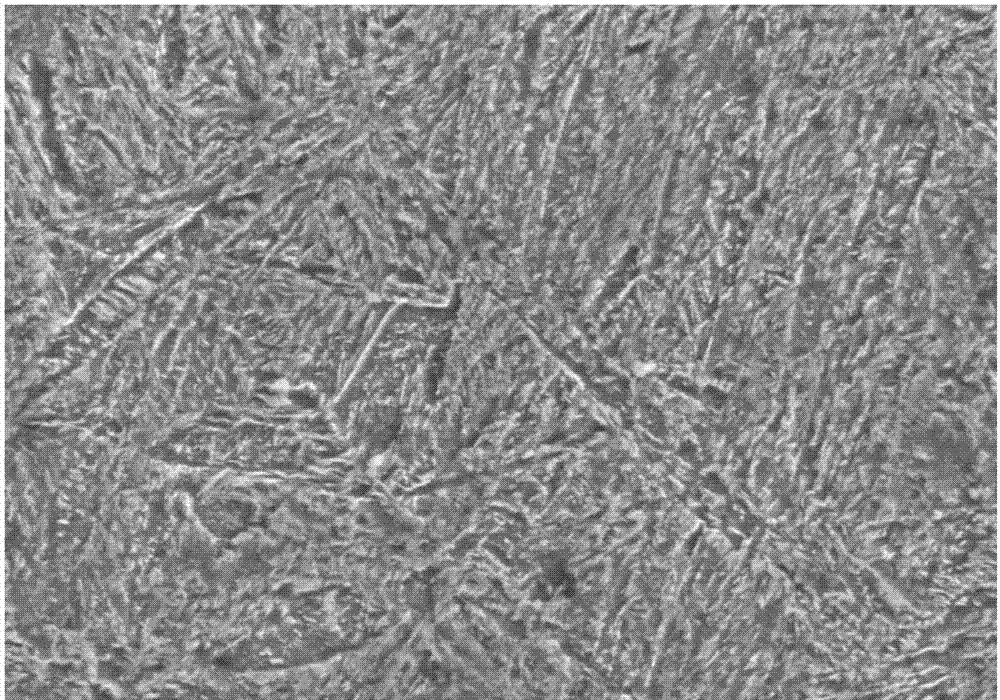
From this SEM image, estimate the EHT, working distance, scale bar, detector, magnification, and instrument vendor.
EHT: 15 kV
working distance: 14.9 mm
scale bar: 8000 nm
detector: SE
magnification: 5 K X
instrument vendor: Hitachi